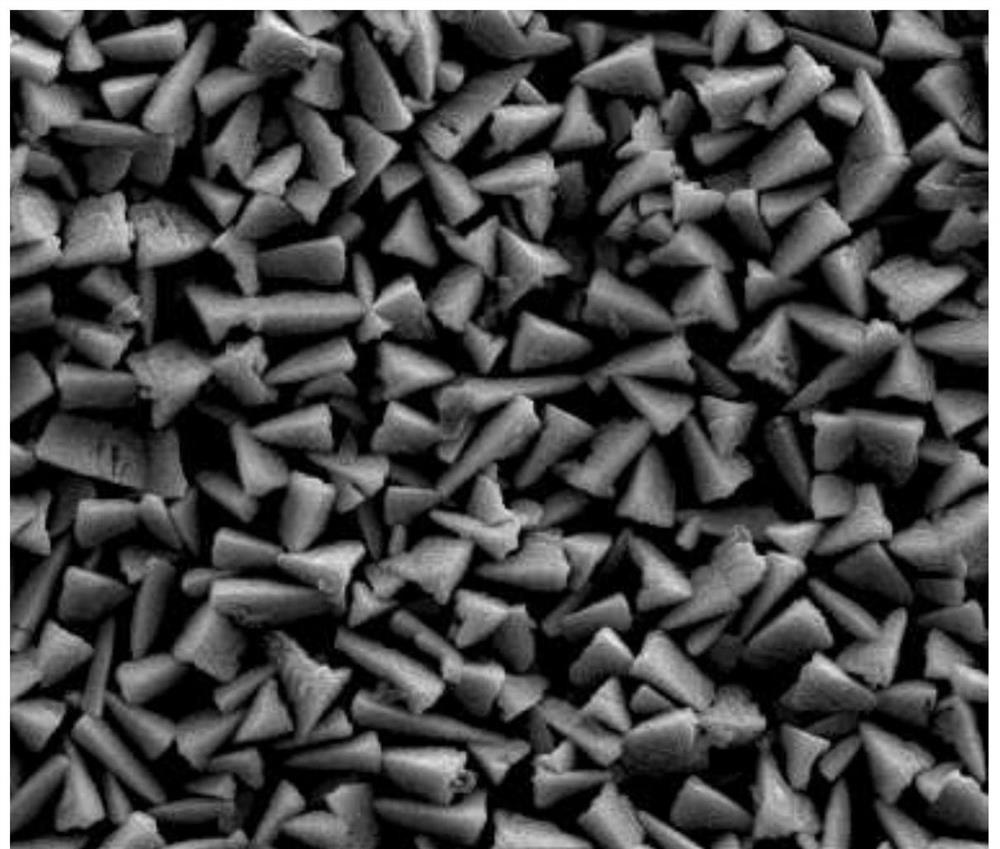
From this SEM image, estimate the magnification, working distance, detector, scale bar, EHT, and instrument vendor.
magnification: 10 K X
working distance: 9.6 mm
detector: SE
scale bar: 10000 nm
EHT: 20 kV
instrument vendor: FEI